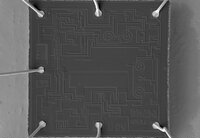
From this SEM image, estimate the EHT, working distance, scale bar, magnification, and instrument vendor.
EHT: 30 kV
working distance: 8.7 mm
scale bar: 1000000 nm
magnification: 0.04 K X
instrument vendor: JEOL